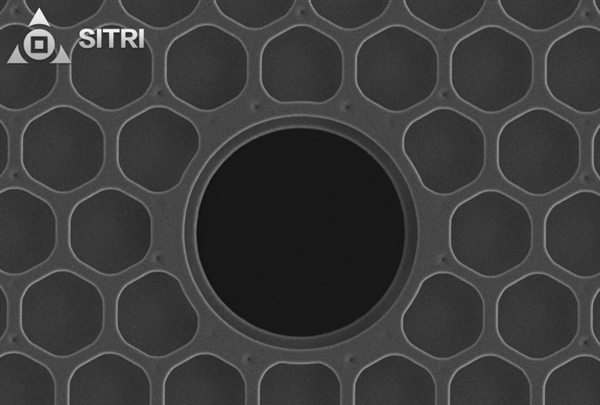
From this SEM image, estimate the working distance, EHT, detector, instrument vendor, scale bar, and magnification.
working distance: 5.1 mm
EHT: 5 kV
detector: SE2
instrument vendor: Zeiss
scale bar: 2000 nm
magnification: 5.8 K X